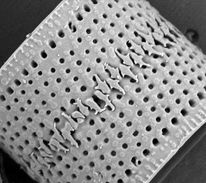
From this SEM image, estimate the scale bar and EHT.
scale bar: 2000 nm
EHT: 2 kV